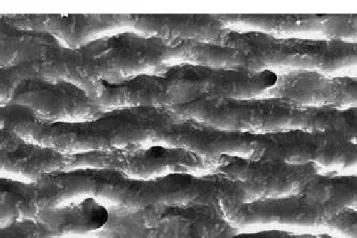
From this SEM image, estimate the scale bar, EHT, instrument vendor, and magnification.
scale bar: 10000 nm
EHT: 15 kV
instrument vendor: JEOL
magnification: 2 K X